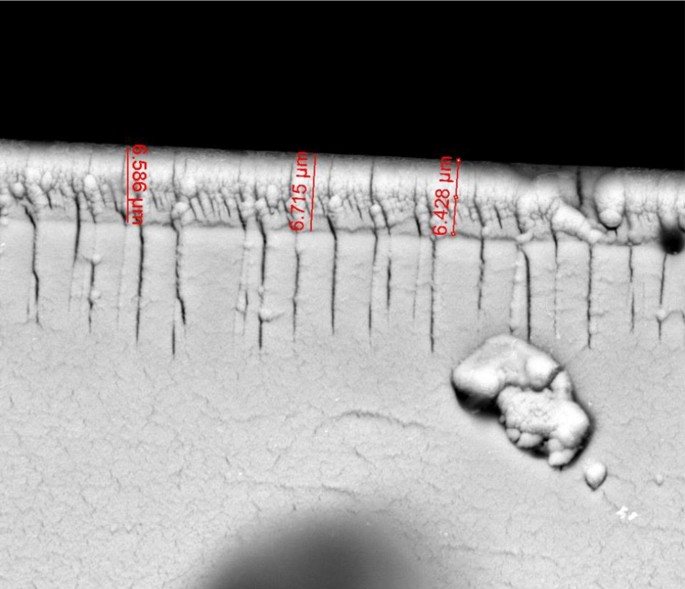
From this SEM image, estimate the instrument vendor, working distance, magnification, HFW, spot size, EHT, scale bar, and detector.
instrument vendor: FEI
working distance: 8.7 mm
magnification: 5 K X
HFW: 59.7 µm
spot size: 4.5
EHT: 30 kV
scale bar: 20000 nm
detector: vCD